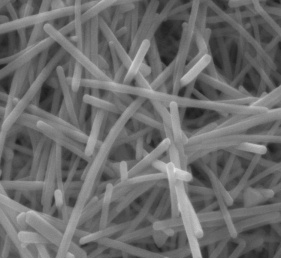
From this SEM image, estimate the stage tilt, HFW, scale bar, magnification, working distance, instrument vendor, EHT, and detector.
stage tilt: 0°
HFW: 2.98 µm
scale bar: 1000 nm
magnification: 100 K X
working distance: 10 mm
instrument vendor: FEI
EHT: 20 kV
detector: ETD